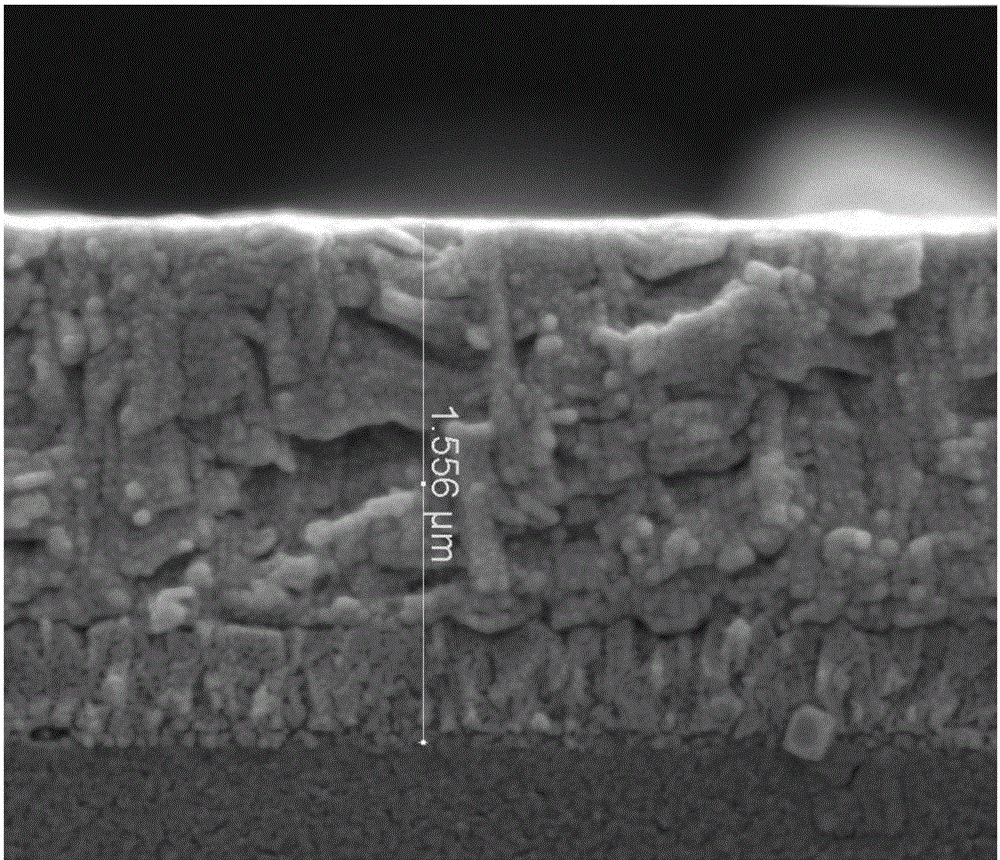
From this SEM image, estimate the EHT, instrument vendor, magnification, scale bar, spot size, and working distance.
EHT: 5 kV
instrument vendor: FEI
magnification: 100 K X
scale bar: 1000 nm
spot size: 3.5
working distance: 8.4 mm